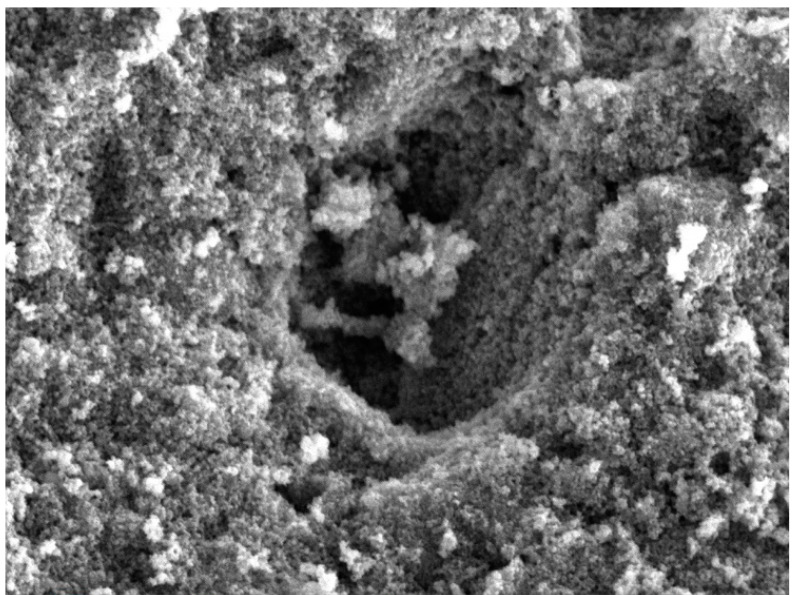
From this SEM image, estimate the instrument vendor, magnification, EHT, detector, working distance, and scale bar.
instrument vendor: Thermo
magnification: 5 K X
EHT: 10 kV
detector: SE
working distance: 10.02 mm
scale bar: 2000 nm